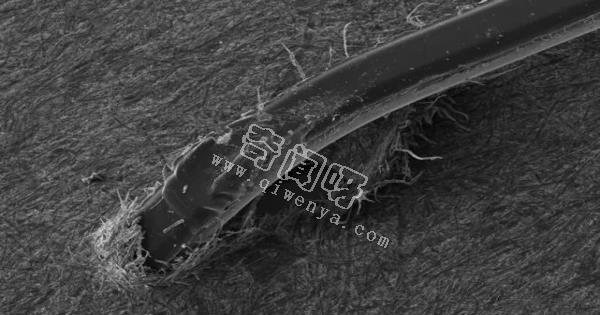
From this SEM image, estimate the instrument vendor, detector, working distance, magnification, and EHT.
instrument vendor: Zeiss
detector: SE2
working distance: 38.9 mm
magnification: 0.03 K X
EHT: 20 kV